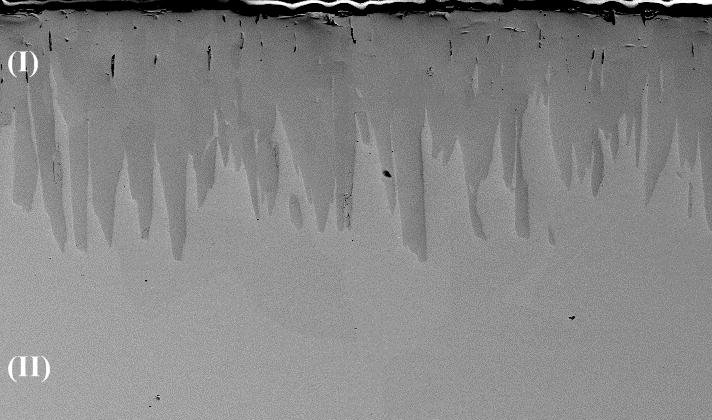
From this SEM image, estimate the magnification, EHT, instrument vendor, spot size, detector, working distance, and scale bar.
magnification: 0.5 K X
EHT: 15 kV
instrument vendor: FEI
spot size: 3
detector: BSE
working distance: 7 mm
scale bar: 100000 nm